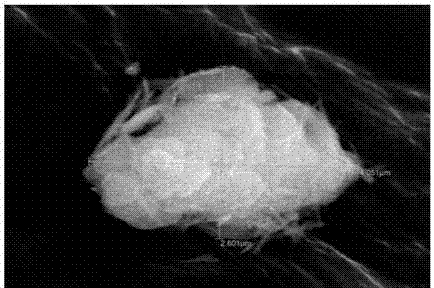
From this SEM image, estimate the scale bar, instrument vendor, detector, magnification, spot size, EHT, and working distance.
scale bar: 1000 nm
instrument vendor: JEOL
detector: SEI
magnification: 20 K X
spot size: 30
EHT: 15 kV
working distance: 21 mm